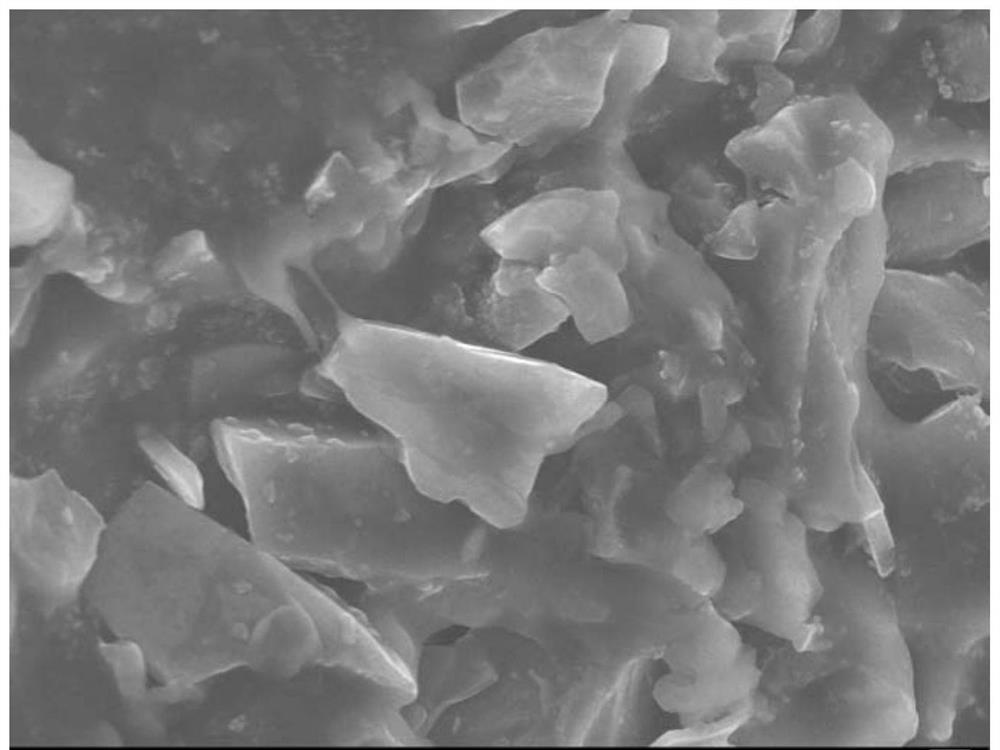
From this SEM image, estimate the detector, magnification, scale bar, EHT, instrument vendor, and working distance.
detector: SEI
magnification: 5 K X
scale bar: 1000 nm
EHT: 10 kV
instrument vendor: JEOL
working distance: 7.8 mm